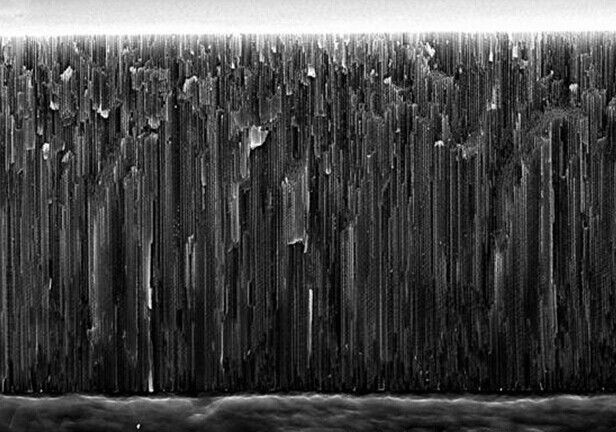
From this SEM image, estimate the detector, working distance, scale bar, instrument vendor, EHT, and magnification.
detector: SE(M)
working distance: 12.7 mm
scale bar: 40000 nm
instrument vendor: Hitachi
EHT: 20 kV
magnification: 1.3 K X